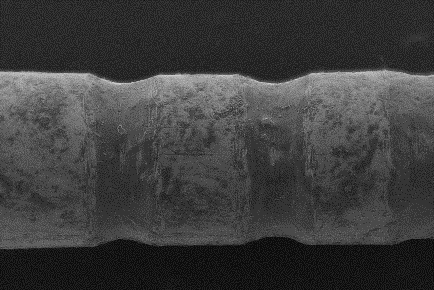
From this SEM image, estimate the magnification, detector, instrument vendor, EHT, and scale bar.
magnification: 0.04 K X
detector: SEI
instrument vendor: JEOL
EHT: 15 kV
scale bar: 500000 nm